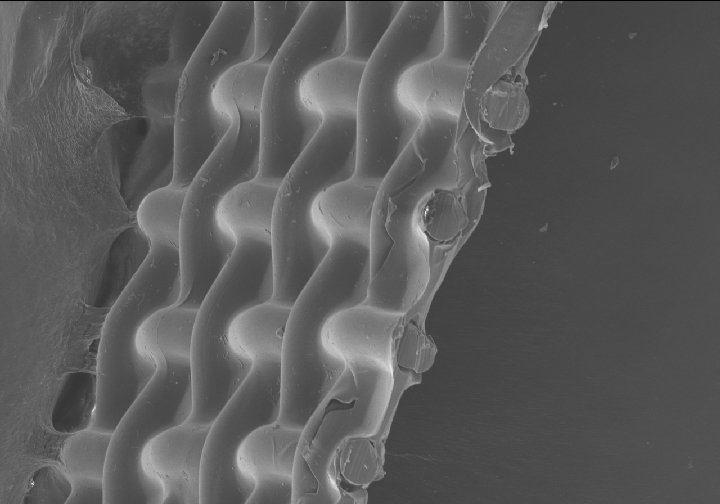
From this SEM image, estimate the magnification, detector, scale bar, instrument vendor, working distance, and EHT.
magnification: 0.25 K X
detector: SE(M)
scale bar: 200000 nm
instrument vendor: Hitachi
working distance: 8.3 mm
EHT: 5 kV